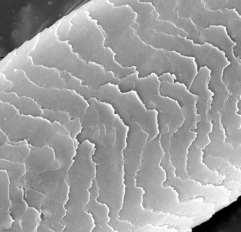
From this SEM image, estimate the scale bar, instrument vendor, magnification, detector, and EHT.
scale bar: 20000 nm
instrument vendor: Tescan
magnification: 2 K X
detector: BE Detector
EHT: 30 kV